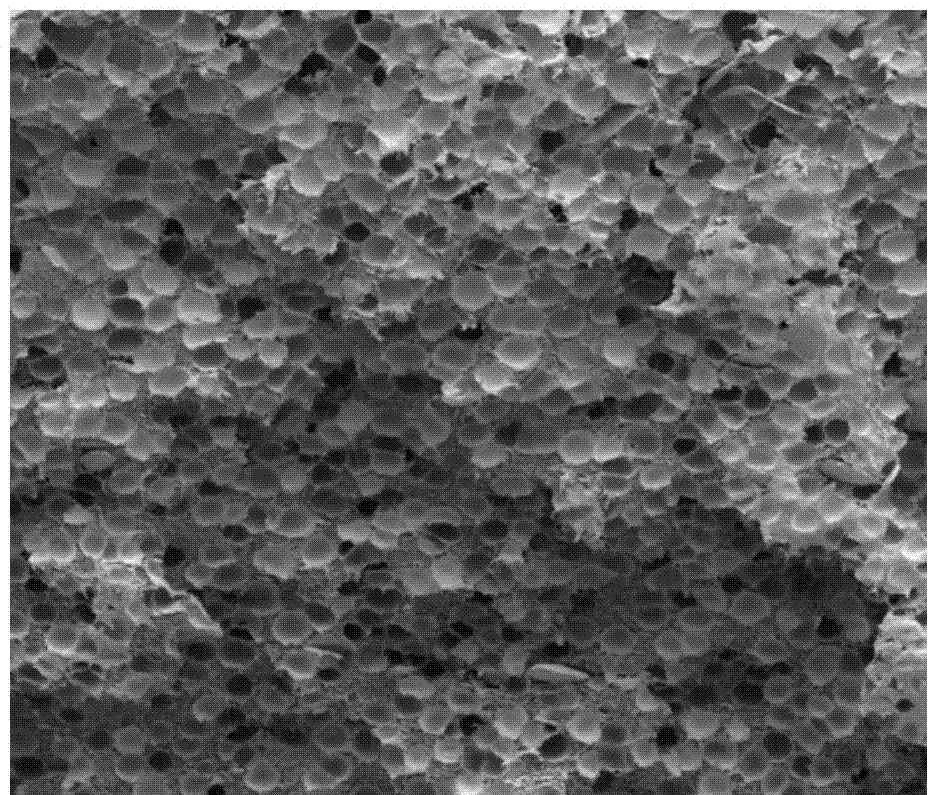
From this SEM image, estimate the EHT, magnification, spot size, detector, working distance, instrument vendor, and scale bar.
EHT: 2 kV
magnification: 0.2 K X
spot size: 2.5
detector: ETD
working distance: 9.2 mm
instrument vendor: FEI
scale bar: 500000 nm